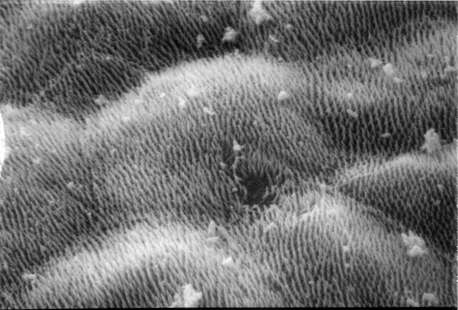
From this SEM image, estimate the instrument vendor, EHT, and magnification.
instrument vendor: JEOL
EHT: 20 kV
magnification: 3 K X